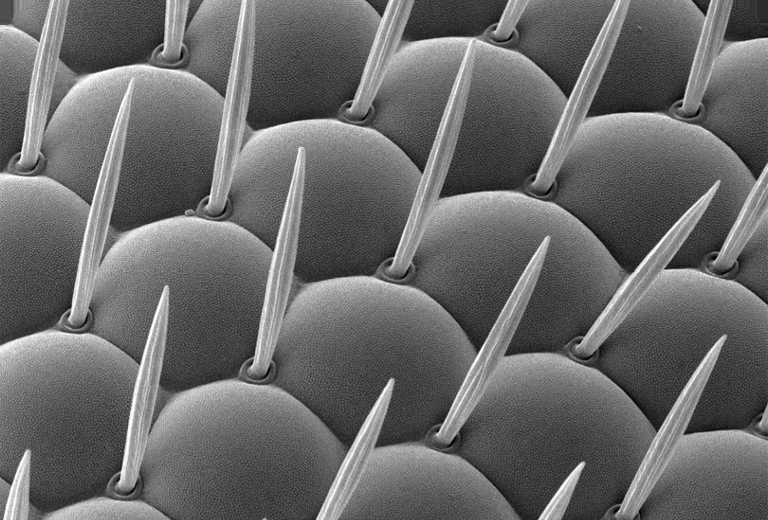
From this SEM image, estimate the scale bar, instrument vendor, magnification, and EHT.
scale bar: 10000 nm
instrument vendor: JEOL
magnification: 1.8 K X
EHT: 10 kV